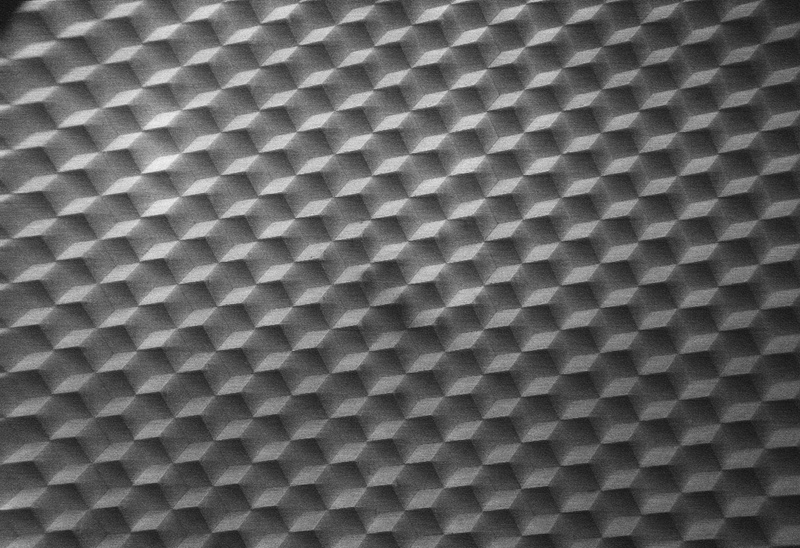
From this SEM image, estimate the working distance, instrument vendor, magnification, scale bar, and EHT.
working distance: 35 mm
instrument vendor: other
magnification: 0.018 K X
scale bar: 100000 nm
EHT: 8.5 kV